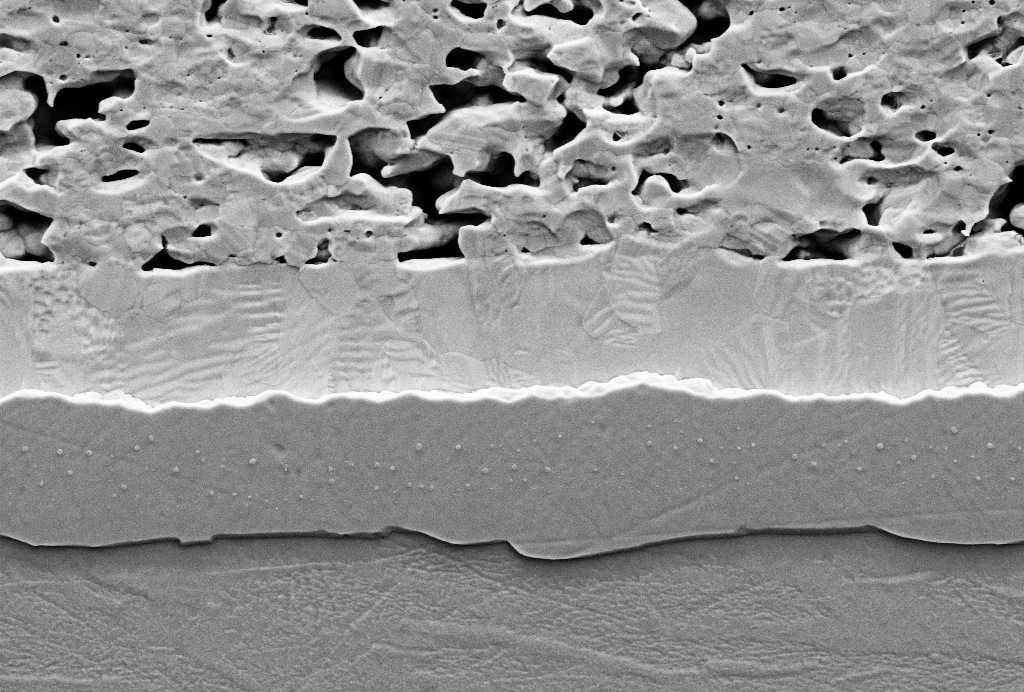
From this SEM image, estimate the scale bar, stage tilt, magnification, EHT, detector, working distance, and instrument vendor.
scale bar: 1000 nm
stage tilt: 0°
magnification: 8 K X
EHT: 5 kV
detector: SE2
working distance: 8 mm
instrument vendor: Zeiss